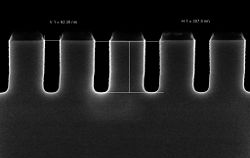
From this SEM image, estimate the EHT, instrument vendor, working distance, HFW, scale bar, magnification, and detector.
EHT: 2 kV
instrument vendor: Zeiss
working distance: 3.2 mm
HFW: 1.701 µm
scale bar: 100 nm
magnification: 200 K X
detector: InLens